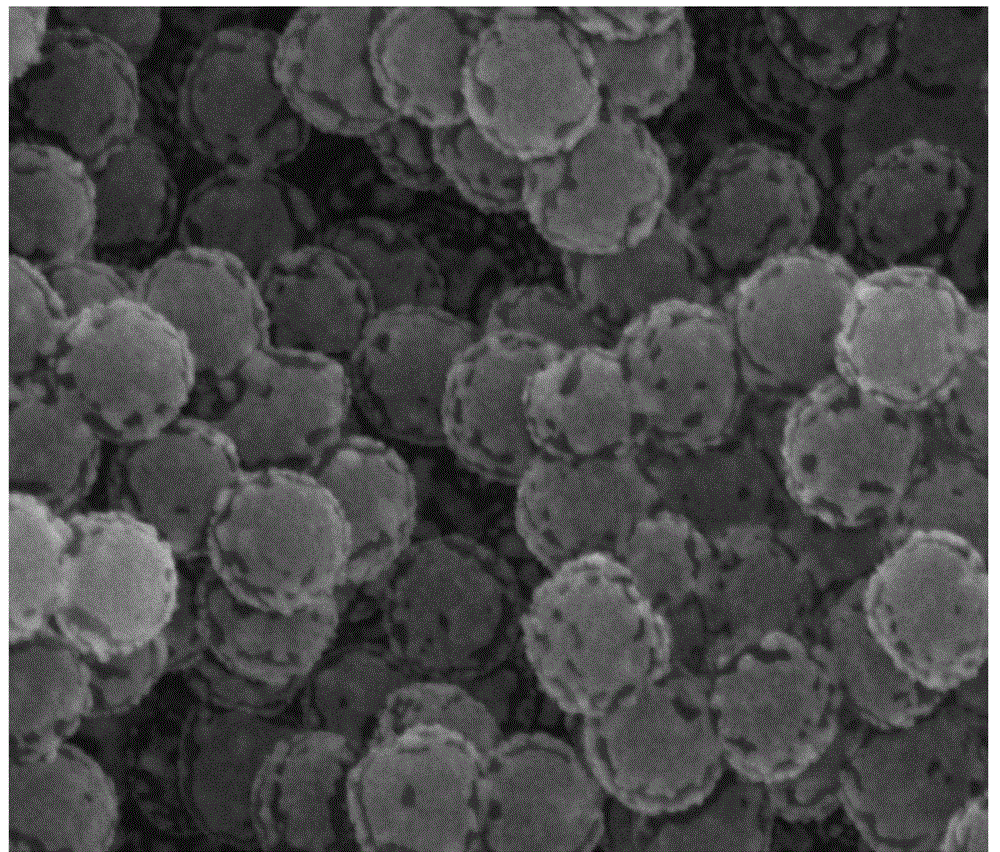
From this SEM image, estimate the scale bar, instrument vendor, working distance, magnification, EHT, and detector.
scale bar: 1000 nm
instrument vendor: FEI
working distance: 9.4 mm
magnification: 100 K X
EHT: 30 kV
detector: SE